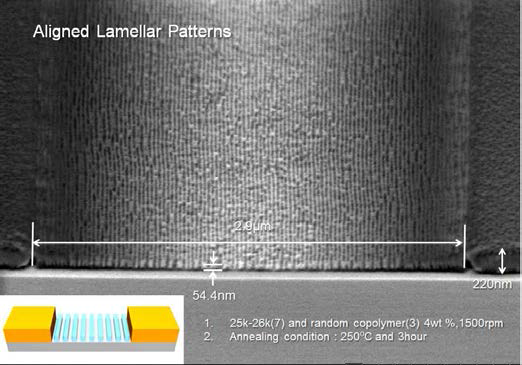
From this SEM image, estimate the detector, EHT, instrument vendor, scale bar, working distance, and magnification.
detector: SE(U)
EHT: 1 kV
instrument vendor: Hitachi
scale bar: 1000 nm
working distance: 4 mm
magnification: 40 K X